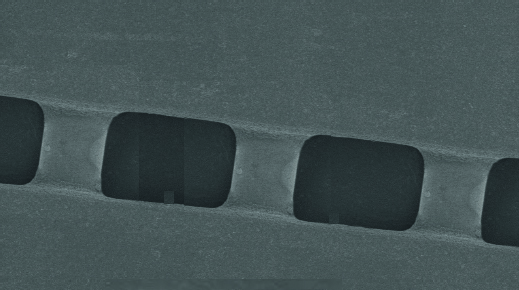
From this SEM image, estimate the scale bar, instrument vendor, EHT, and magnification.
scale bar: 50000 nm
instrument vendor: JEOL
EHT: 17 kV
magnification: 0.5 K X